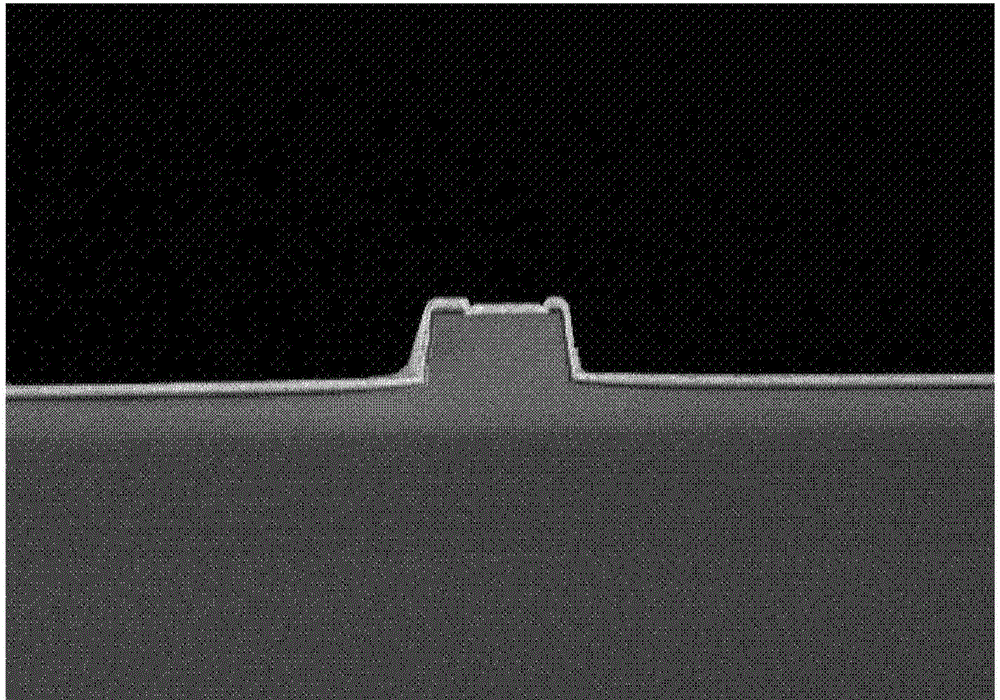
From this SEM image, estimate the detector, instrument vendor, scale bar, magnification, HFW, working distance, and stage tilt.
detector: TLD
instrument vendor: FEI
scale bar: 10000 nm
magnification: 8 K X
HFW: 37.3 µm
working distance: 5.9 mm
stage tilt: -4°